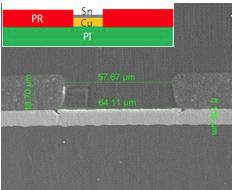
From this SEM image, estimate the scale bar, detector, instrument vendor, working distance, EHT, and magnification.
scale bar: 50000 nm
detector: SE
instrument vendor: FEI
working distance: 13.1 mm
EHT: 10 kV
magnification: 2.4 K X